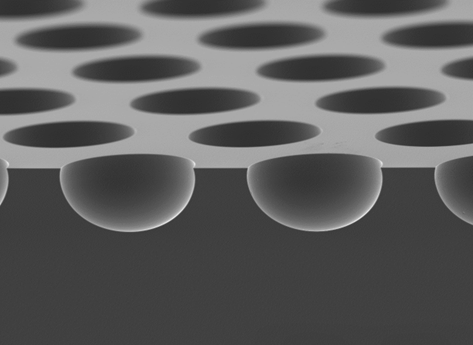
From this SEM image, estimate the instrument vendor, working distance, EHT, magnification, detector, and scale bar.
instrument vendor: JEOL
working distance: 9.6 mm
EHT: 5 kV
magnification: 1 K X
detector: LED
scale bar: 10000 nm